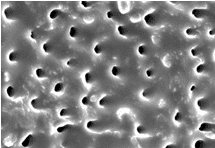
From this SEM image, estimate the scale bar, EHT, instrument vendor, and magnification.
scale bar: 10000 nm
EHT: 10 kV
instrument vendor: JEOL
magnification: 2.5 K X